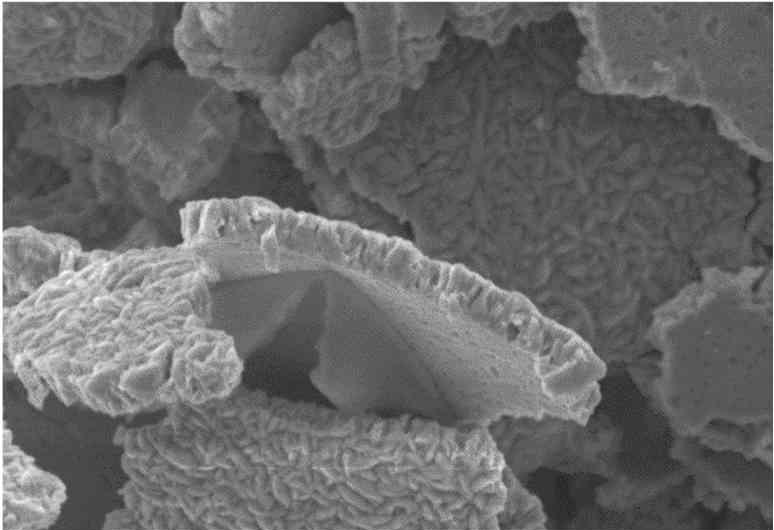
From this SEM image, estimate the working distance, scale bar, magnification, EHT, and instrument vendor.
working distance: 16 mm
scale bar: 5000 nm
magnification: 5 K X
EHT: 10 kV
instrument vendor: Zeiss